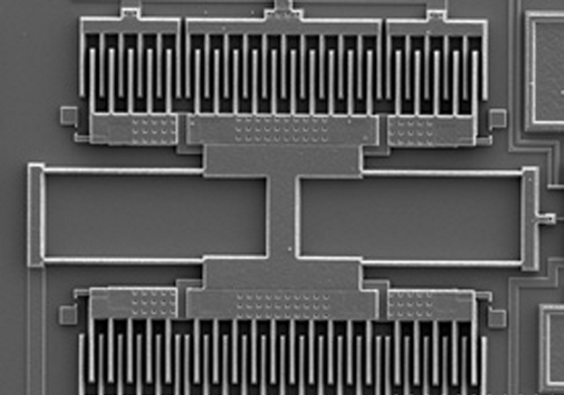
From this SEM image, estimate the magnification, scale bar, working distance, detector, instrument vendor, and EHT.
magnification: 0.35 K X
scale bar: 100000 nm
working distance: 14.8 mm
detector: SE(U)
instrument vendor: Hitachi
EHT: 10 kV